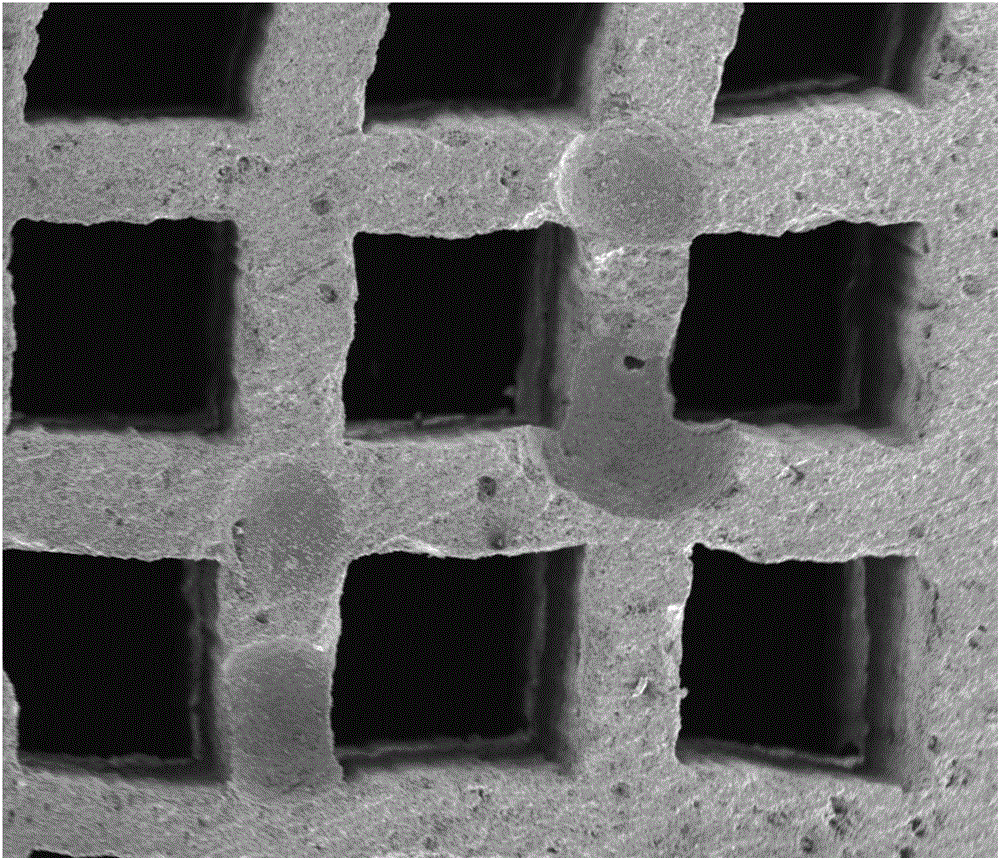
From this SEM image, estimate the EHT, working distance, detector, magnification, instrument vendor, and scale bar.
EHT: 15 kV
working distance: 4.9 mm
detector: ETD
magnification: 0.15 K X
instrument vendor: FEI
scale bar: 500000 nm